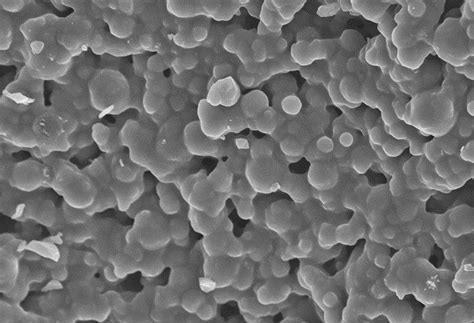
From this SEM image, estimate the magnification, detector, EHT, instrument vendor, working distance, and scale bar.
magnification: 10 K X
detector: SE(M)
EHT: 5 kV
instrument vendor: Hitachi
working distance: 8.9 mm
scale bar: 5000 nm